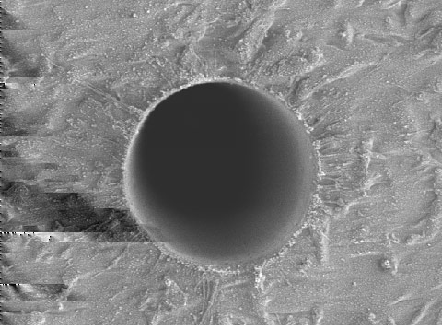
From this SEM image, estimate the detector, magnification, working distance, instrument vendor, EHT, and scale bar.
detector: SEI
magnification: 1 K X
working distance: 11 mm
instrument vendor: JEOL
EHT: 3 kV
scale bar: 10000 nm